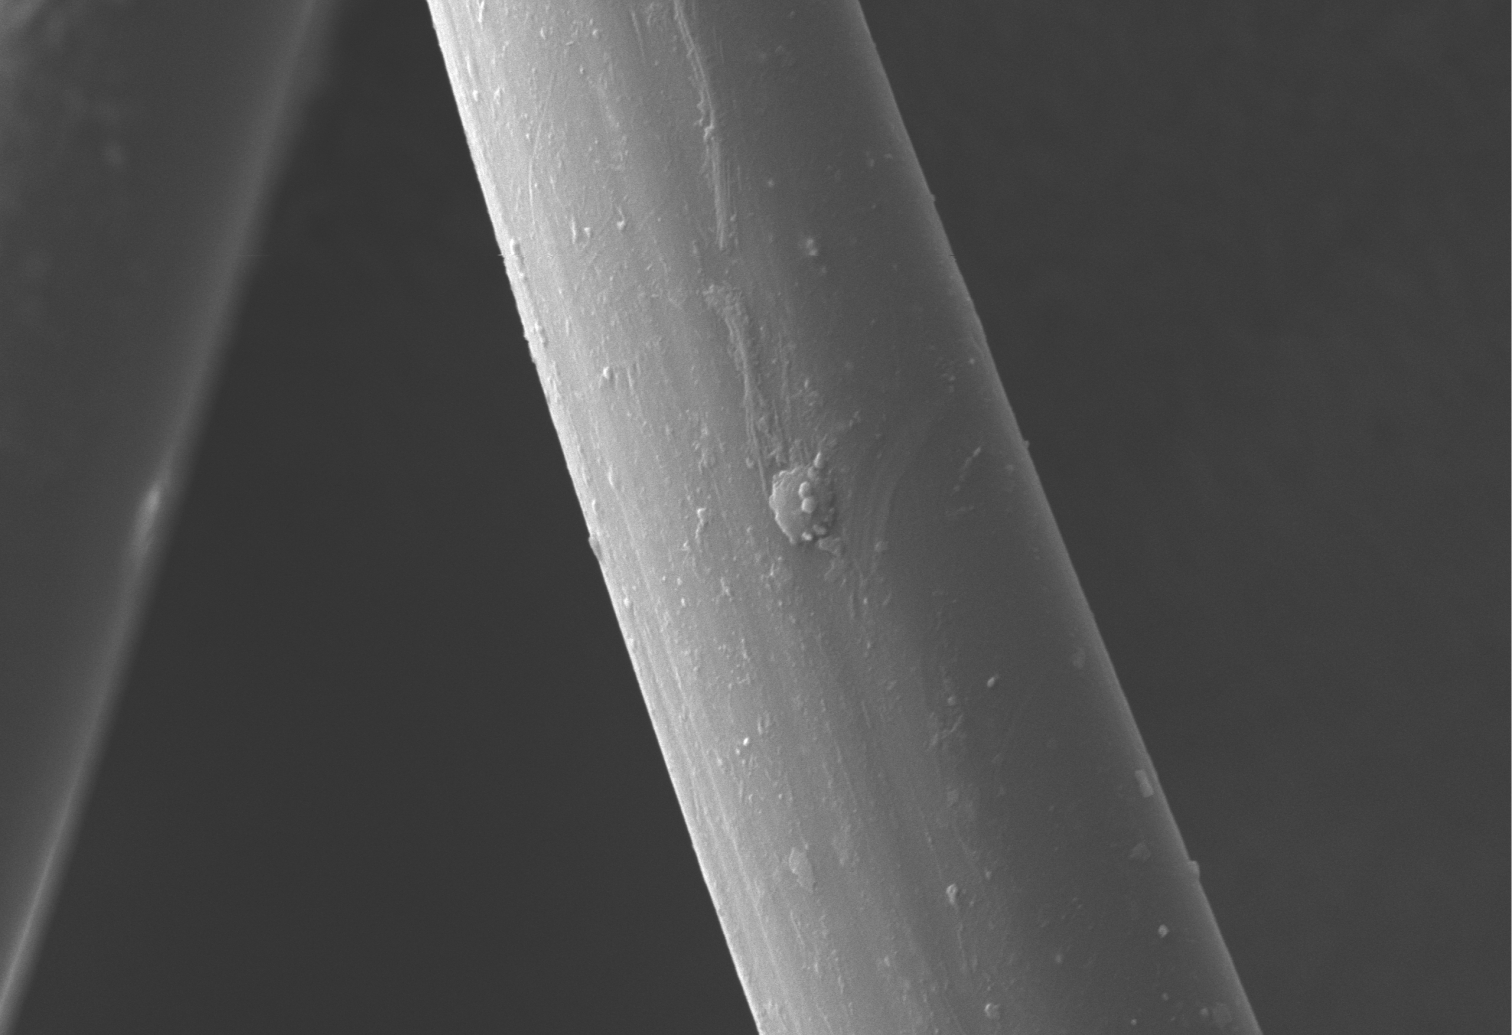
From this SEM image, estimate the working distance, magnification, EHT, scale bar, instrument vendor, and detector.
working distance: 10 mm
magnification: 2 K X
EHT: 10 kV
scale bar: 10000 nm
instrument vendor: JEOL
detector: SEI